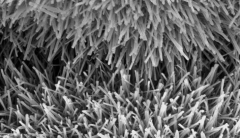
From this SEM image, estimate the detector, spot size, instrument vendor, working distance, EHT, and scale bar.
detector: SE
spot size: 2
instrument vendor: FEI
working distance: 9 mm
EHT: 20 kV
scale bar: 5000 nm